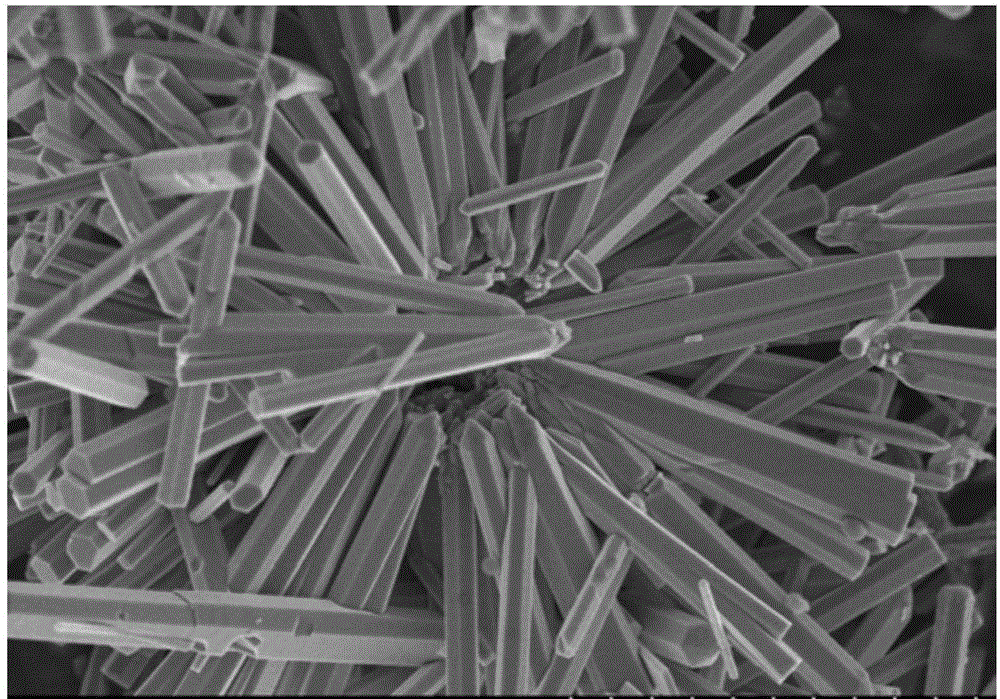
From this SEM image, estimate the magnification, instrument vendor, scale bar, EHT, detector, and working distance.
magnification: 13 K X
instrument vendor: Hitachi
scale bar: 4000 nm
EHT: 3 kV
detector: SE(M)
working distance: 8.8 mm